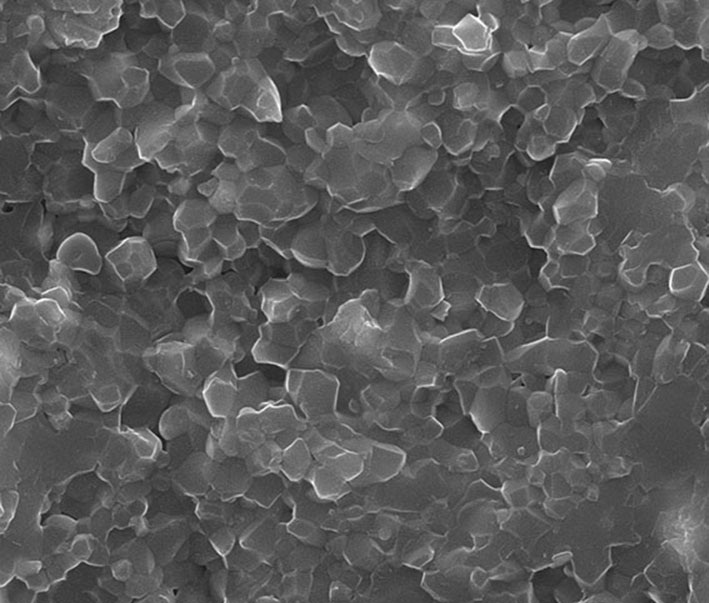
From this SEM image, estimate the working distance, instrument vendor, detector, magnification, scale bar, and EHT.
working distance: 12.1 mm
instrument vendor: FEI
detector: SE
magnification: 50 K X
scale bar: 2000 nm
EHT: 20 kV